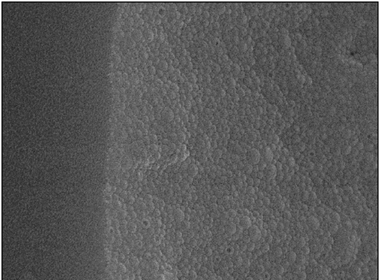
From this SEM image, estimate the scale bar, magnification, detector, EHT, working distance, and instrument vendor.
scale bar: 1000 nm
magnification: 20 K X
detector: SEI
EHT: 15 kV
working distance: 6.1 mm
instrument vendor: JEOL